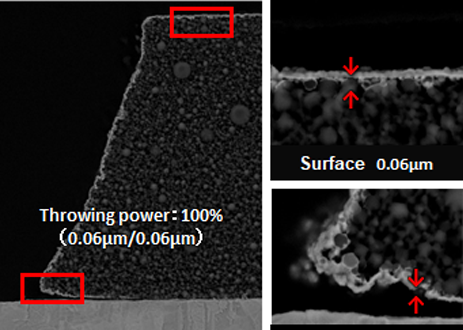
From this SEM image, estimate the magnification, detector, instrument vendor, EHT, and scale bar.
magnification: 5 K X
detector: BED-C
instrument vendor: JEOL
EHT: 5 kV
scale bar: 1000 nm